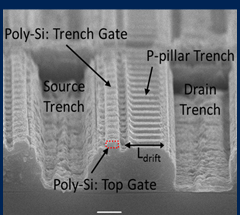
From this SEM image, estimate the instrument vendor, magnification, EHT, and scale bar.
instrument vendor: JEOL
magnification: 6 K X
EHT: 20 kV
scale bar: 2000 nm